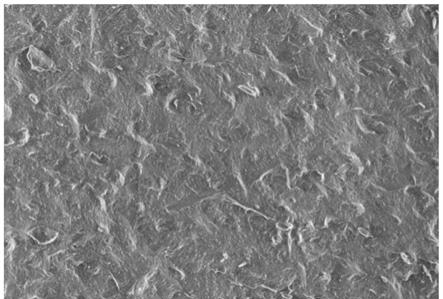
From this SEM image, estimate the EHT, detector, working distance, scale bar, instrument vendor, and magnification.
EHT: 1 kV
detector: SE(UL)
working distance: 8.4 mm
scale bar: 5000 nm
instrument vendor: Hitachi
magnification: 10 K X